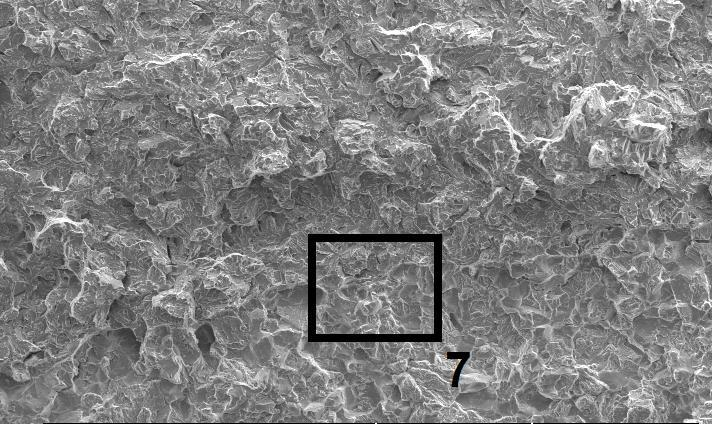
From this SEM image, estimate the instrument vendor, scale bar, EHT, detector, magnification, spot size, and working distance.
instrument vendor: FEI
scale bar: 200000 nm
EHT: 20 kV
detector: SE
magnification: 0.1 K X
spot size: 5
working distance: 10.5 mm